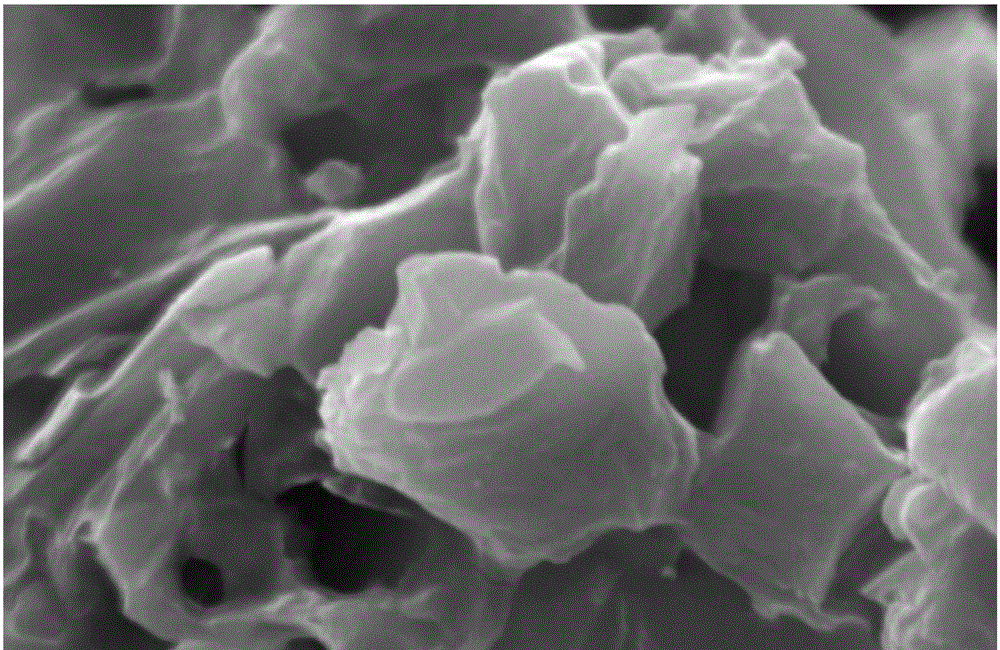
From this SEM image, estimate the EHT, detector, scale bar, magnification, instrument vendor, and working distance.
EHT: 10 kV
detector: SE1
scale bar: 1000 nm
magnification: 10 K X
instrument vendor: Zeiss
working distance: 5.5 mm